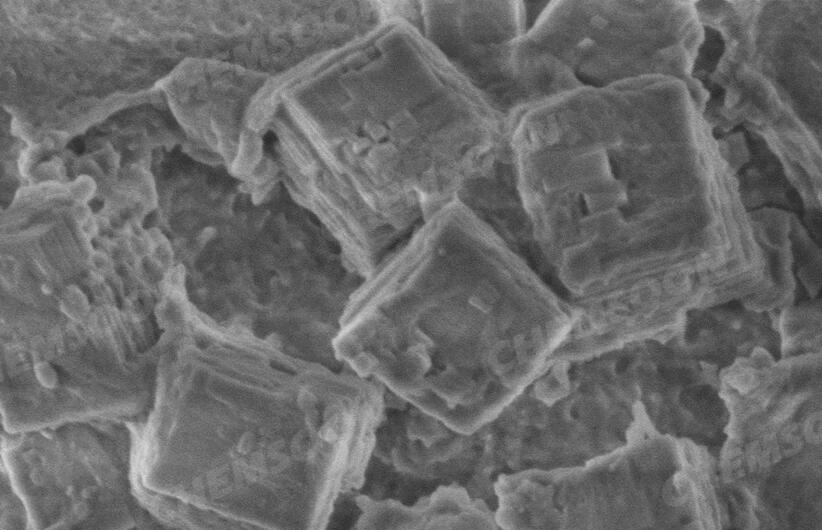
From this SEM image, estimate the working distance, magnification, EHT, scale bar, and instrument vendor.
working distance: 3.4 mm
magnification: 70 K X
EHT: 1 kV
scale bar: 500 nm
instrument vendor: Hitachi